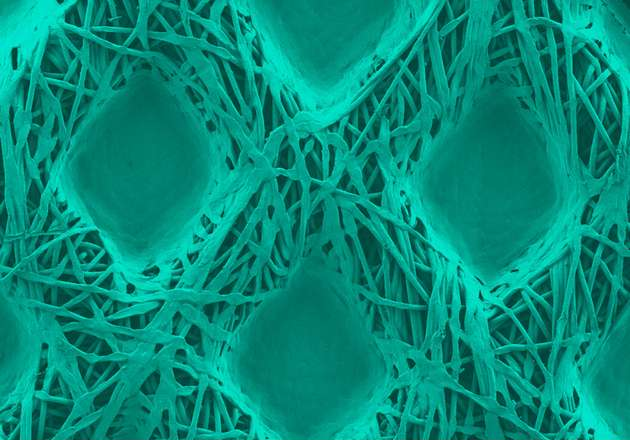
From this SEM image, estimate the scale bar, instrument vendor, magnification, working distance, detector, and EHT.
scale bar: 1000000 nm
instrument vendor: Hitachi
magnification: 0.03 K X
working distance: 8.2 mm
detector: SE(M)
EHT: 2 kV